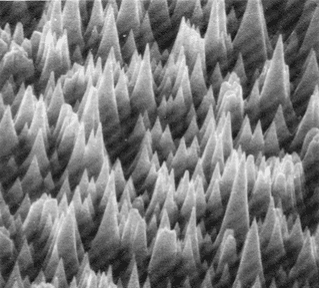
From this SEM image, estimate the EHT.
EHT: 30 kV